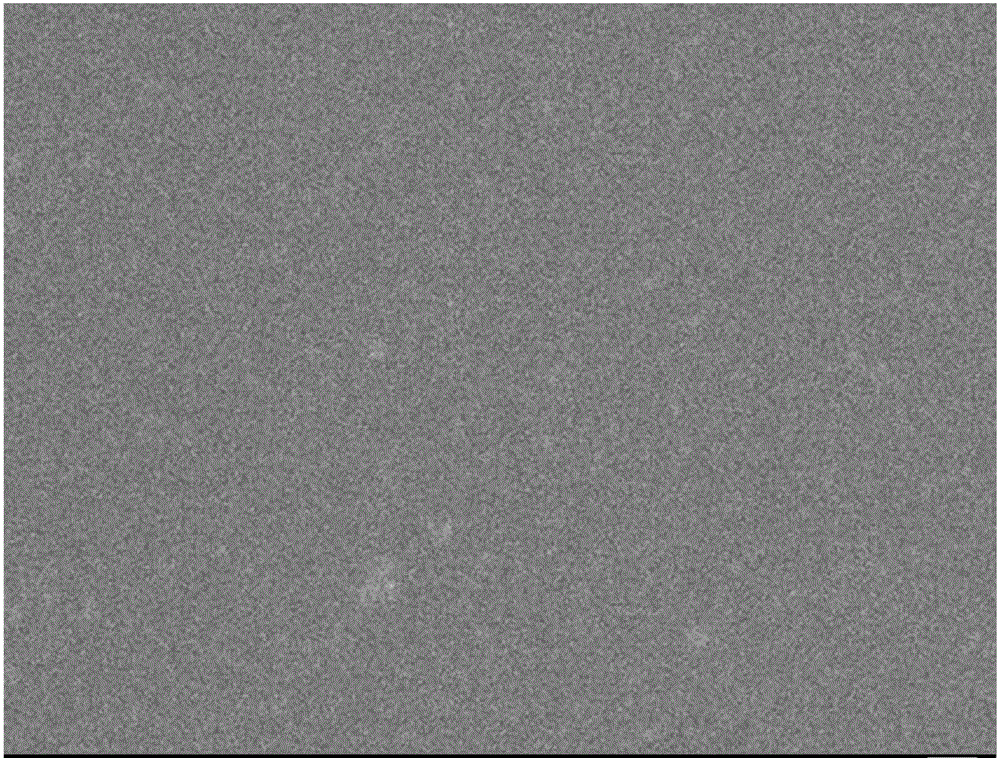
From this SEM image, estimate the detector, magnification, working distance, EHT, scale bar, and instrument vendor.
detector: SEI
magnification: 30 K X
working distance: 7.1 mm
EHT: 5 kV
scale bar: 100 nm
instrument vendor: JEOL